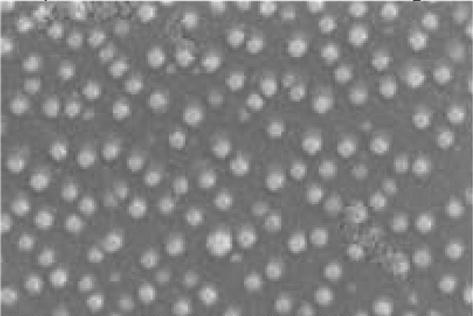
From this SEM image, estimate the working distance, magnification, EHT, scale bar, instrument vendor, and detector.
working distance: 4 mm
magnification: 100 K X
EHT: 5 kV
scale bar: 100 nm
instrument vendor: Zeiss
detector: InLens